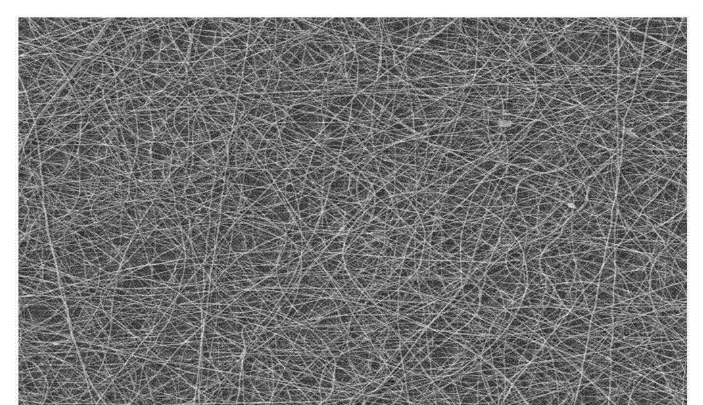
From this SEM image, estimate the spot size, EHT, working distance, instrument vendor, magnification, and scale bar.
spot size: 3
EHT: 20 kV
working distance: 7.3 mm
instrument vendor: FEI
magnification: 1 K X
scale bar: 100000 nm